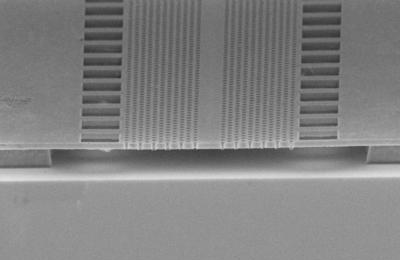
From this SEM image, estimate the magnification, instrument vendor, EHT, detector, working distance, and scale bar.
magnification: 5.11 K X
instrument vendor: Zeiss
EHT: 5 kV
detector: InLens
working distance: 6 mm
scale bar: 1000 nm